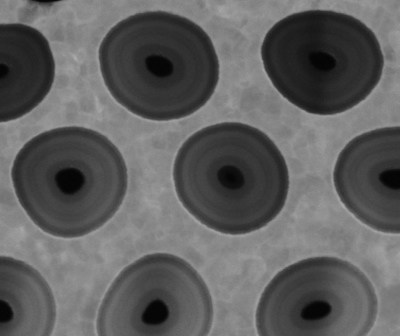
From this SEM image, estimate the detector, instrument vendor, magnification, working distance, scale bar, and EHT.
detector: STEM II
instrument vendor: FEI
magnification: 650 K X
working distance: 4.4 mm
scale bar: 100 nm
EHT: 30 kV